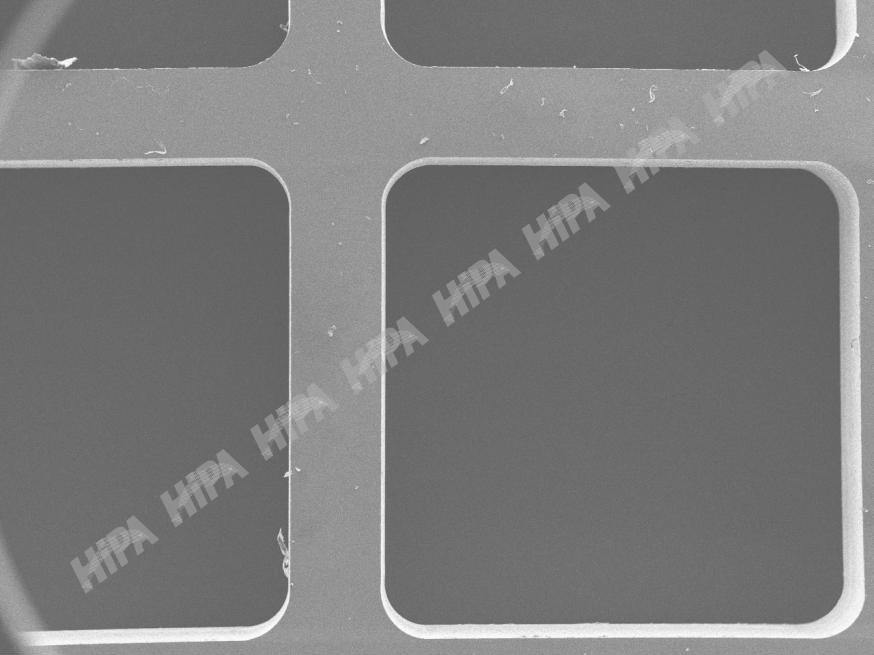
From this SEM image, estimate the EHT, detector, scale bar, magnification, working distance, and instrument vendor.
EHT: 15 kV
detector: SED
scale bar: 500000 nm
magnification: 0.03 K X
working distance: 12.9 mm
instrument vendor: JEOL